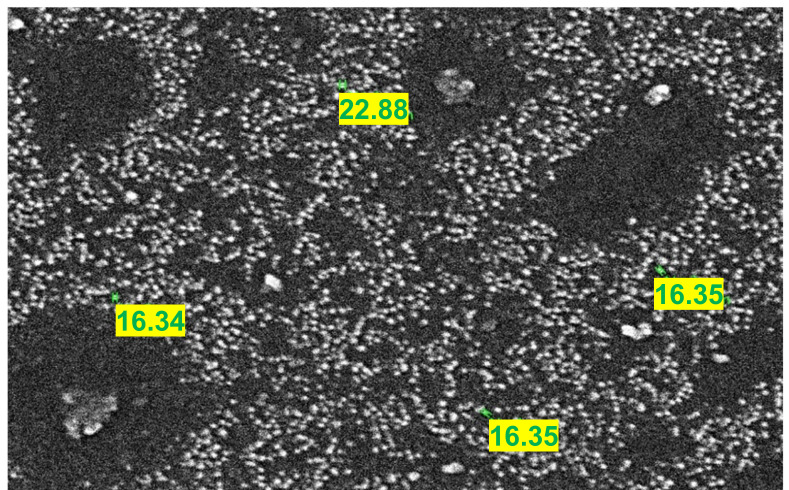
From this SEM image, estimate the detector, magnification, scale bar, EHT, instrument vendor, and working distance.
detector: SED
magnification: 40 K X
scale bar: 500 nm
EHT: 20 kV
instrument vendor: JEOL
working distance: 16.1 mm